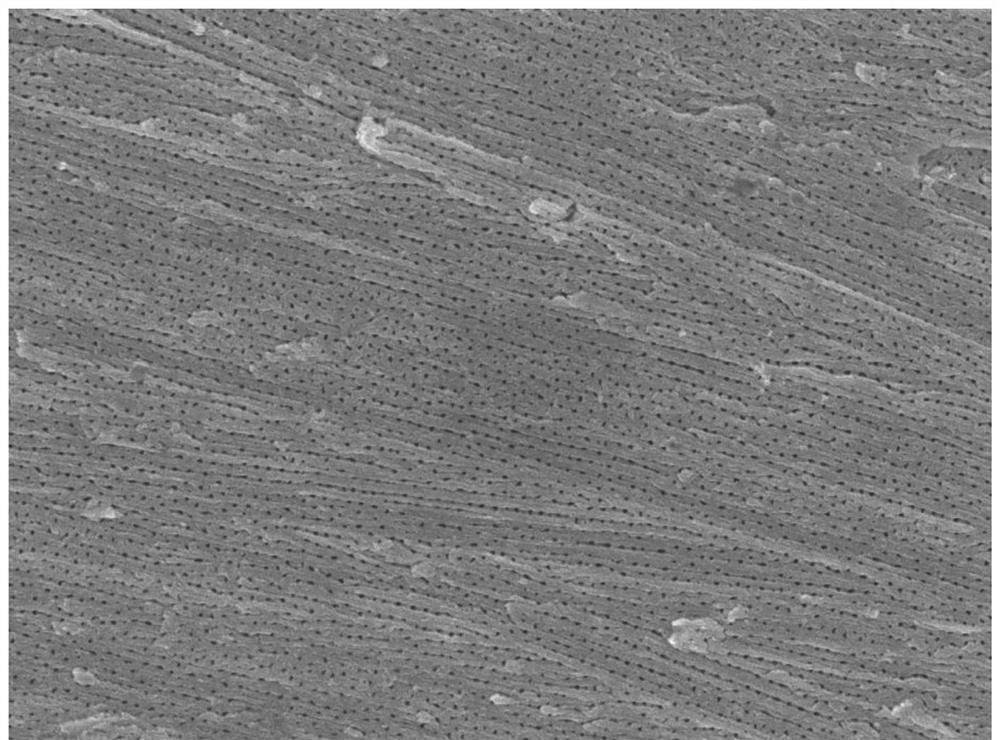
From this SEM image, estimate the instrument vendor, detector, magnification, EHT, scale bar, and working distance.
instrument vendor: JEOL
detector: SEI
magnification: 20 K X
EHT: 5 kV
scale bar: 1000 nm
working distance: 7.9 mm